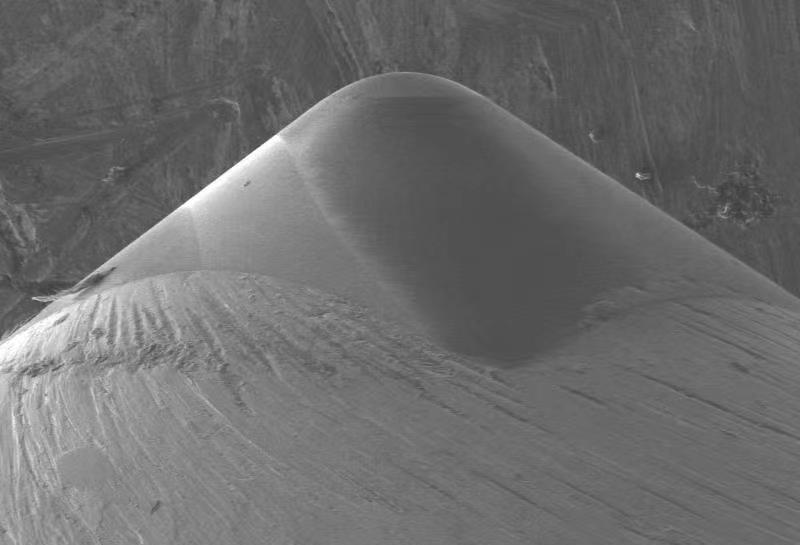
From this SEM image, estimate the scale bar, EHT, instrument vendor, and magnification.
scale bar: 500000 nm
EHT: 10 kV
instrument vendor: Hitachi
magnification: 0.1 K X